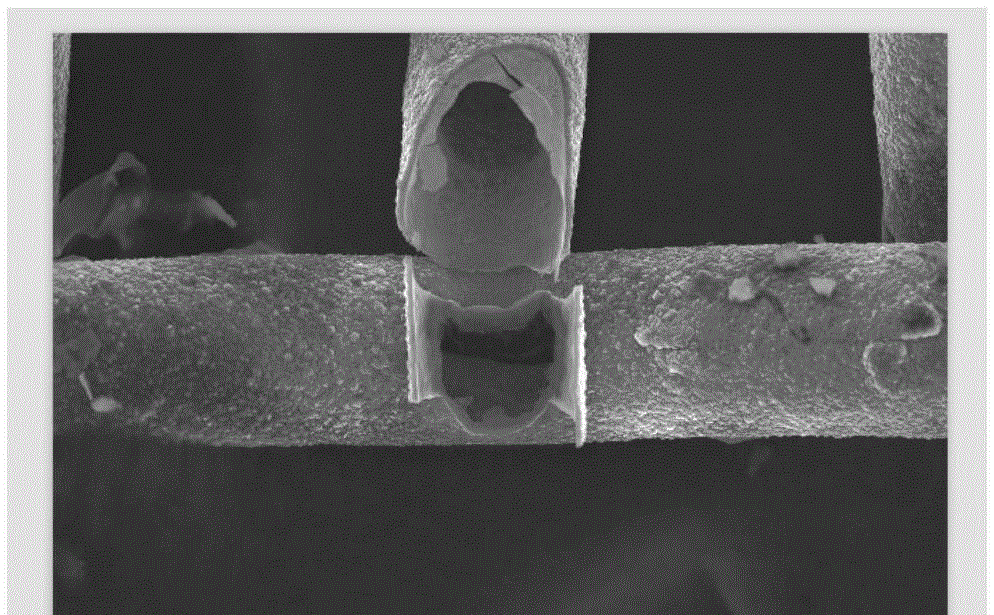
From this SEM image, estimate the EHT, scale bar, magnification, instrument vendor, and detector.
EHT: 25 kV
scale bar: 50000 nm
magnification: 0.5 K X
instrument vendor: JEOL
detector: SEI